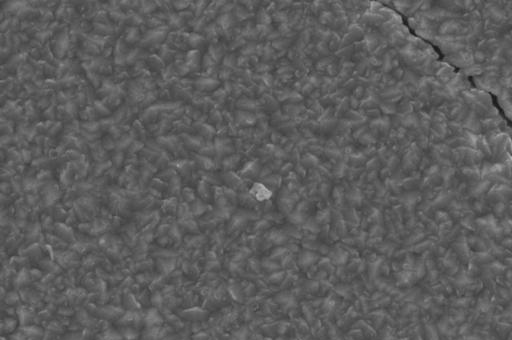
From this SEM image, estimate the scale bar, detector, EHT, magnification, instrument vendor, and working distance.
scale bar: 1000 nm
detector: SE1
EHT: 15 kV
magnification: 8 K X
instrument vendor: Zeiss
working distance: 18 mm